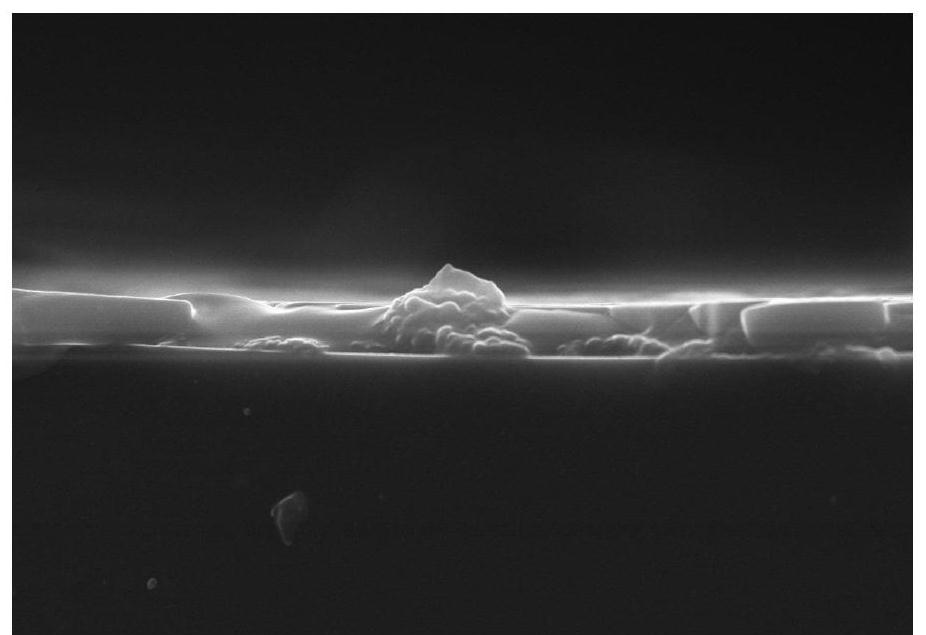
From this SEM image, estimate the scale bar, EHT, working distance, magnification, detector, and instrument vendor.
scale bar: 5000 nm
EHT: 10 kV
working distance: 7.8 mm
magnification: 10 K X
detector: SE(M)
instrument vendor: Hitachi